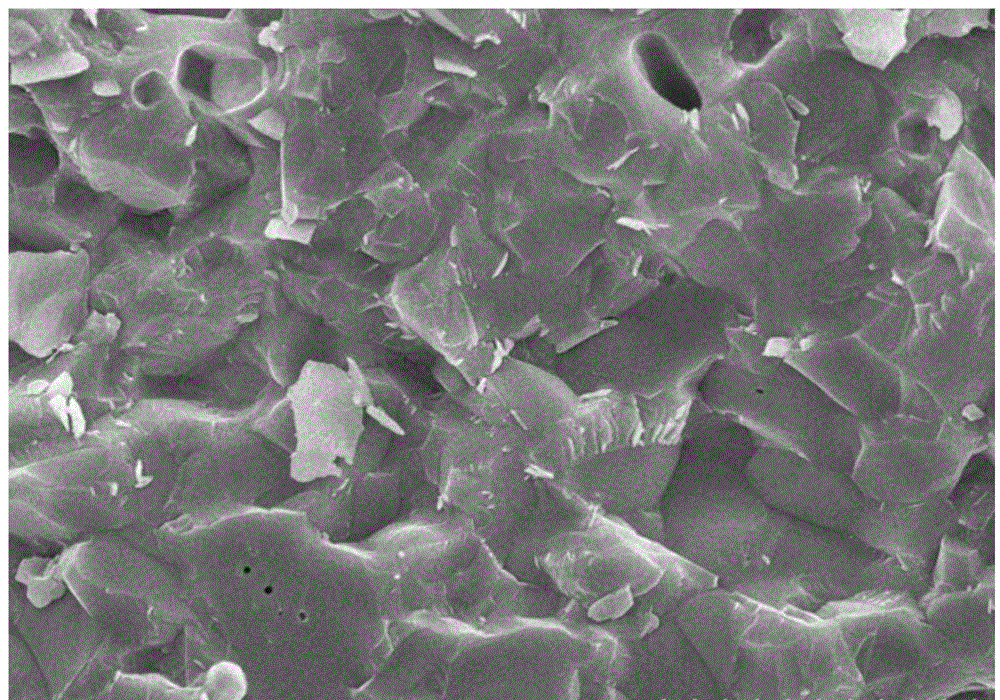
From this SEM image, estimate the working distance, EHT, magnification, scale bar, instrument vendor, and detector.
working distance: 5.3 mm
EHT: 15 kV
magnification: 4 K X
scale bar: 10000 nm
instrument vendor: Hitachi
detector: SE